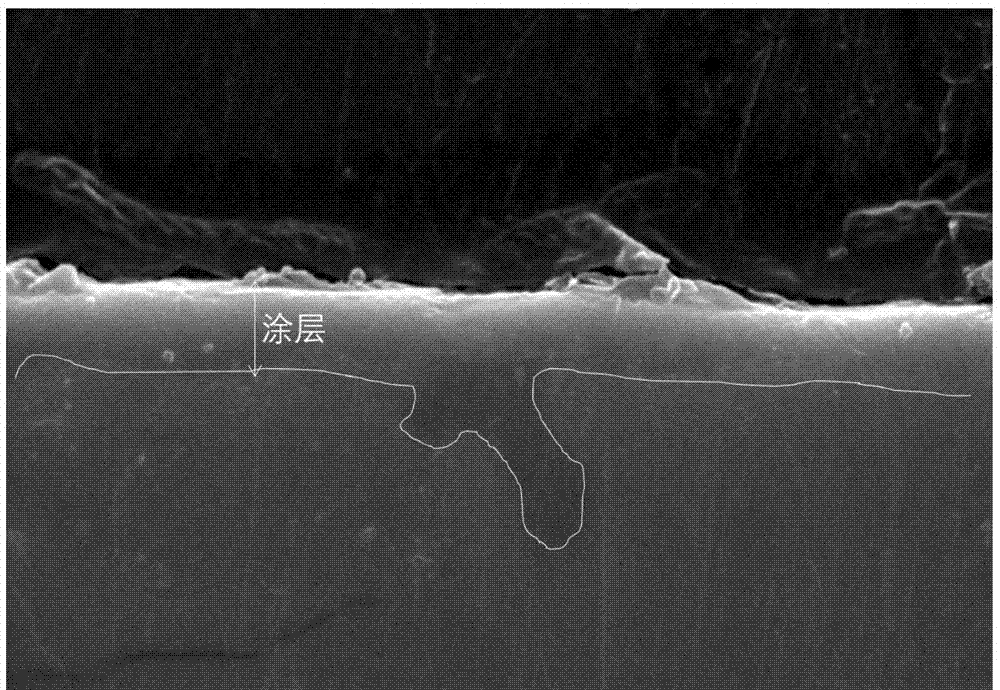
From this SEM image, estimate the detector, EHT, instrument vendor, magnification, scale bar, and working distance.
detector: SE(U,LA0)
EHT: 20 kV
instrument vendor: Hitachi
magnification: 8 K X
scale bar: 5000 nm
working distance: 14.9 mm